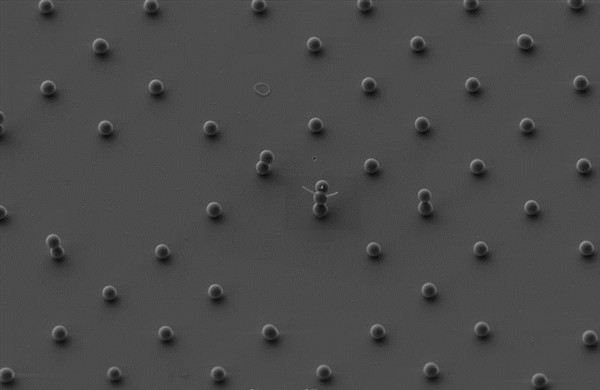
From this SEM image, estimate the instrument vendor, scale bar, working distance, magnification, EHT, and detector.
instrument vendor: Zeiss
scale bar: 2000 nm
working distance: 4.4 mm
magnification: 3 K X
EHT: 3 kV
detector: SE2